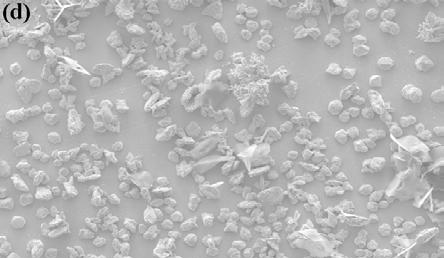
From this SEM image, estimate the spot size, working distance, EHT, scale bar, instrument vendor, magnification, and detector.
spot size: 6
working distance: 12.1 mm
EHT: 20 kV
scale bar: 200000 nm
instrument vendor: FEI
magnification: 0.1 K X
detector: SE